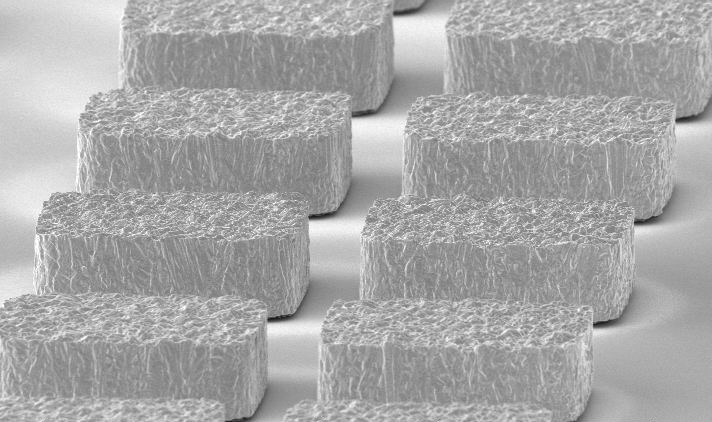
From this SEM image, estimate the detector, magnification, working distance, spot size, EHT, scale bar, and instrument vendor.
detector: SE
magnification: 1 K X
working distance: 9.1 mm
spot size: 3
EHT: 10 kV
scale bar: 20000 nm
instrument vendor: FEI